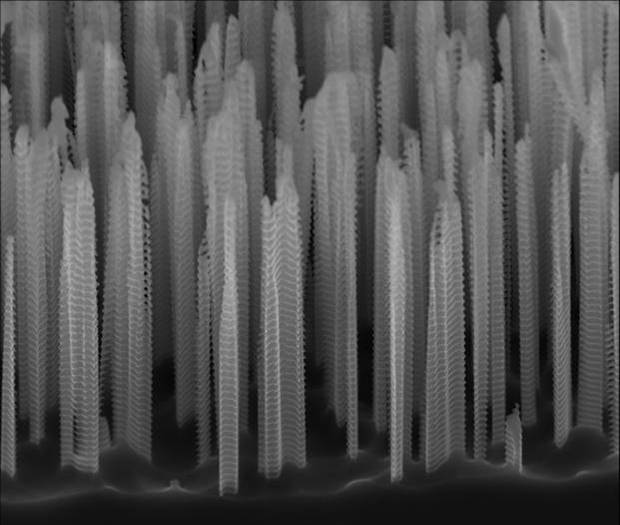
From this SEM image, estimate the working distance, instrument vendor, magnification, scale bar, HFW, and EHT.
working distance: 4.8 mm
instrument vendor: FEI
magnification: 8 K X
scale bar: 5000 nm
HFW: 16 µm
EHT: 10 kV